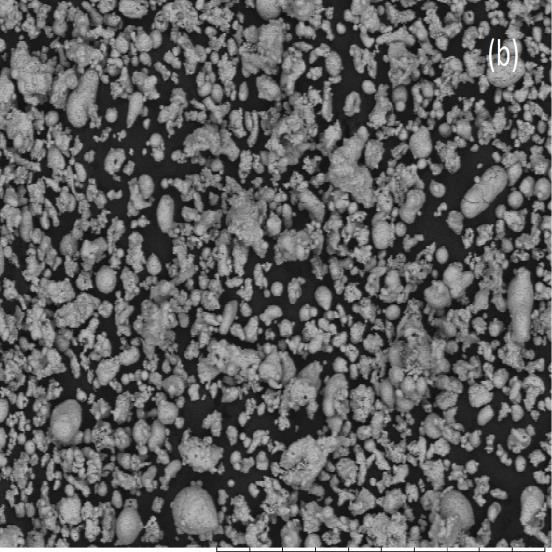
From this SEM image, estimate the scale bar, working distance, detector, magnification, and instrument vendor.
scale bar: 500000 nm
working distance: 6.2 mm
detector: HL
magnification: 0.2 K X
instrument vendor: Hitachi